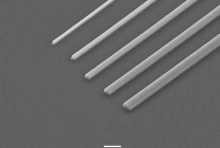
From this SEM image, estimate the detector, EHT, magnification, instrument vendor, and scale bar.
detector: SEI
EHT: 20 kV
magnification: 1 K X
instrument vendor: JEOL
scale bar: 10000 nm